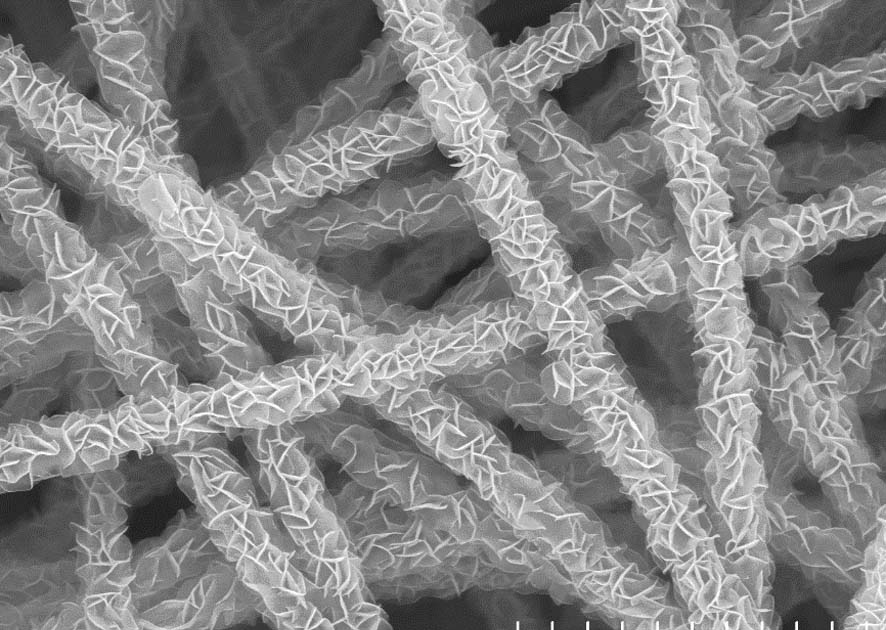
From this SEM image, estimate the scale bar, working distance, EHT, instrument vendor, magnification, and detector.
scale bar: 5000 nm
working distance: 12.8 mm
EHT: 15 kV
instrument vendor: Hitachi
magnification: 10 K X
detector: SE(U)